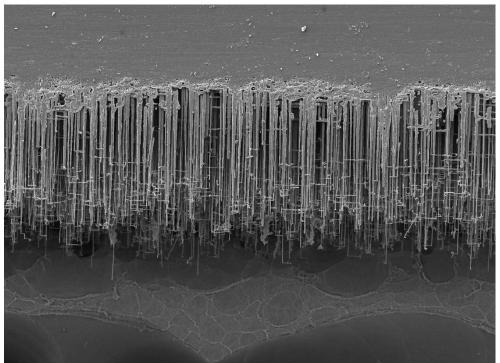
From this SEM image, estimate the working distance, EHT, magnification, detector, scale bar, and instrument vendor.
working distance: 10.1 mm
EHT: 5 kV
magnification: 0.6 K X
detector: LED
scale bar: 10000 nm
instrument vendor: JEOL